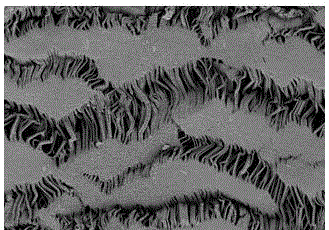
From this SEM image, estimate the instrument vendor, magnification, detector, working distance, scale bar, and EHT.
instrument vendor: Zeiss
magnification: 2 K X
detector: SE2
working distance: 5.3 mm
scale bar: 10000 nm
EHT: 1 kV